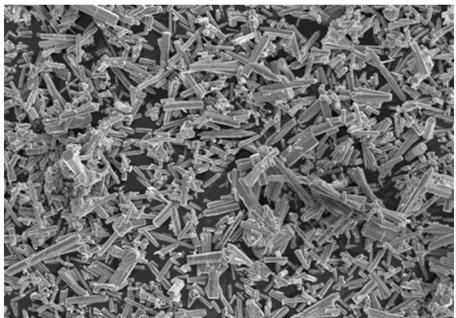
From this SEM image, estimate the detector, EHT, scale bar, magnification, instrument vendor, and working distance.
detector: SE(M)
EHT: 10 kV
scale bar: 50000 nm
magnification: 1 K X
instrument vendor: Hitachi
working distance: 13.6 mm